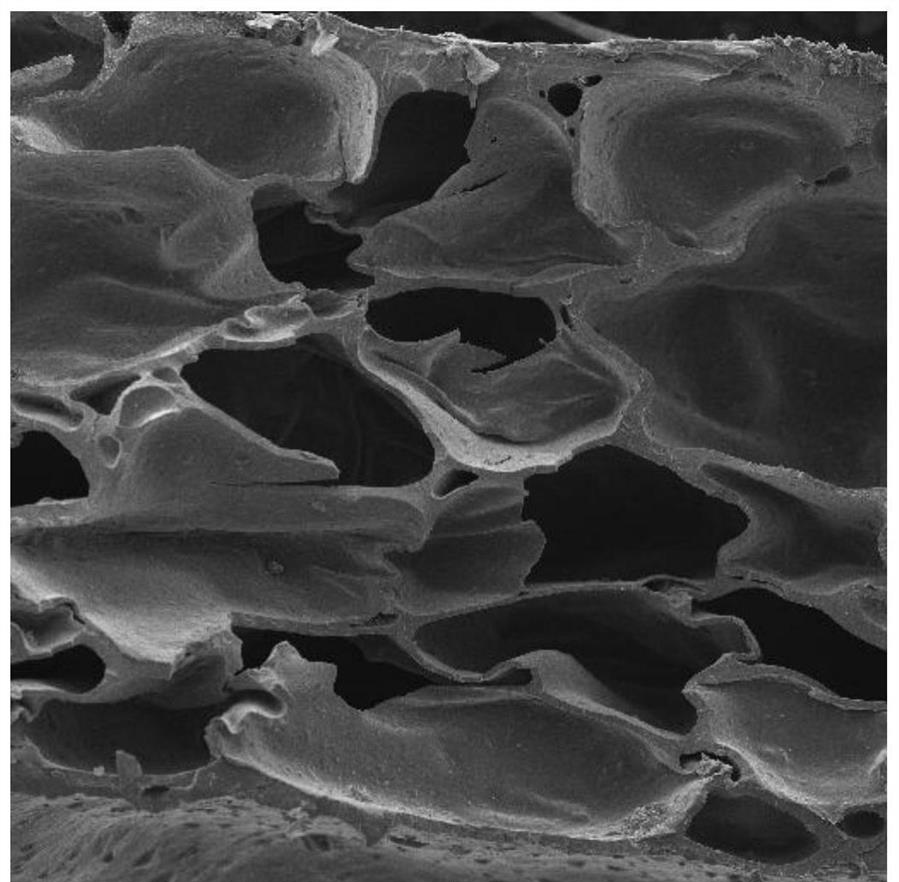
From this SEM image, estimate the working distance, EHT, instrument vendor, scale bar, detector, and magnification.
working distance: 8.33 mm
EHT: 7 kV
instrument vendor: Tescan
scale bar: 200000 nm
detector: SE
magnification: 0.15 K X